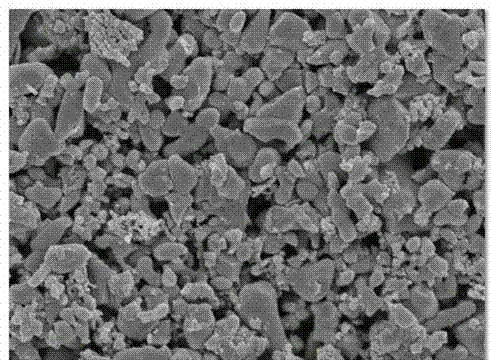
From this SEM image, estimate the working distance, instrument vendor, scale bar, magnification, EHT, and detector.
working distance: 8.7 mm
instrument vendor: JEOL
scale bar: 1000 nm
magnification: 20 K X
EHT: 5 kV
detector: SEI